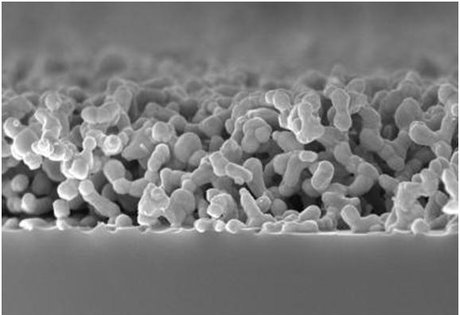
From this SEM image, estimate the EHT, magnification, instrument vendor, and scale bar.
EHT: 10 kV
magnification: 18 K X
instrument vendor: Hitachi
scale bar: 3000 nm